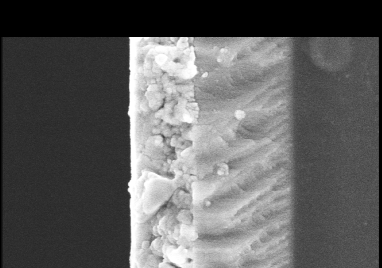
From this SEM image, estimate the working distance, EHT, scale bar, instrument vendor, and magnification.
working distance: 12 mm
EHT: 9 kV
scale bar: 1000 nm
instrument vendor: JEOL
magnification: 30 K X